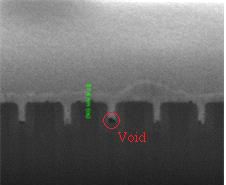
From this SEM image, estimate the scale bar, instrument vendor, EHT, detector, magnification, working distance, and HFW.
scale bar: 500 nm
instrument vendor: FEI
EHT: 5 kV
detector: ETD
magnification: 200 K X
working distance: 10 mm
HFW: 1.49 µm